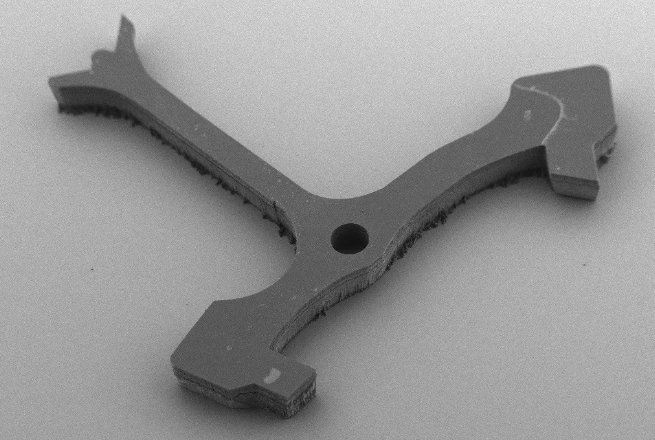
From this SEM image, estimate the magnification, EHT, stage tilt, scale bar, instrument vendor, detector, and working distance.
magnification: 0.044 K X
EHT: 3 kV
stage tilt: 40°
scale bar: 100000 nm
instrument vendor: Zeiss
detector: HE-SE2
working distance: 7.3 mm